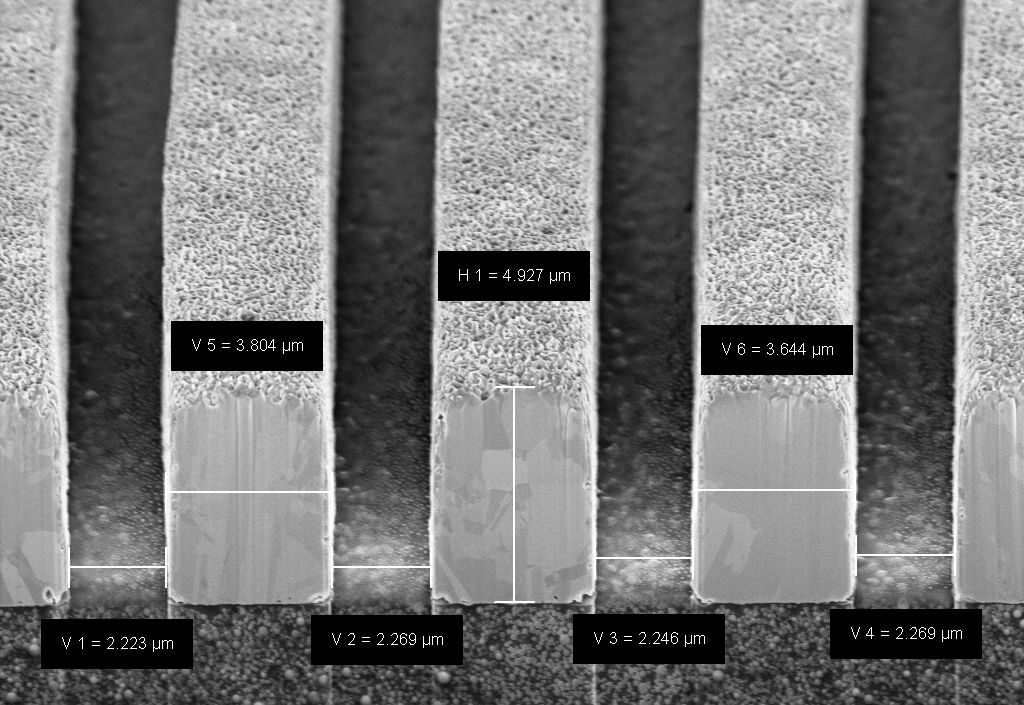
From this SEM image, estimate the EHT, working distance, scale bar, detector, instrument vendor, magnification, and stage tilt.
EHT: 5 kV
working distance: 5.3 mm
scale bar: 2000 nm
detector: InLens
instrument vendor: Zeiss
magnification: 5 K X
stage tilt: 54.1°